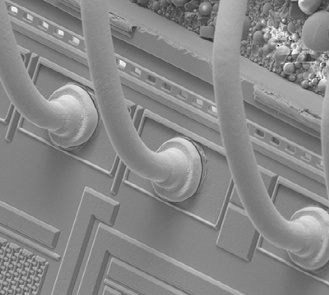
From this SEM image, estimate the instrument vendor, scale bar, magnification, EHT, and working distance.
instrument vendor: FEI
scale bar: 100000 nm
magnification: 1.2 K X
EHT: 10 kV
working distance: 5.8 mm